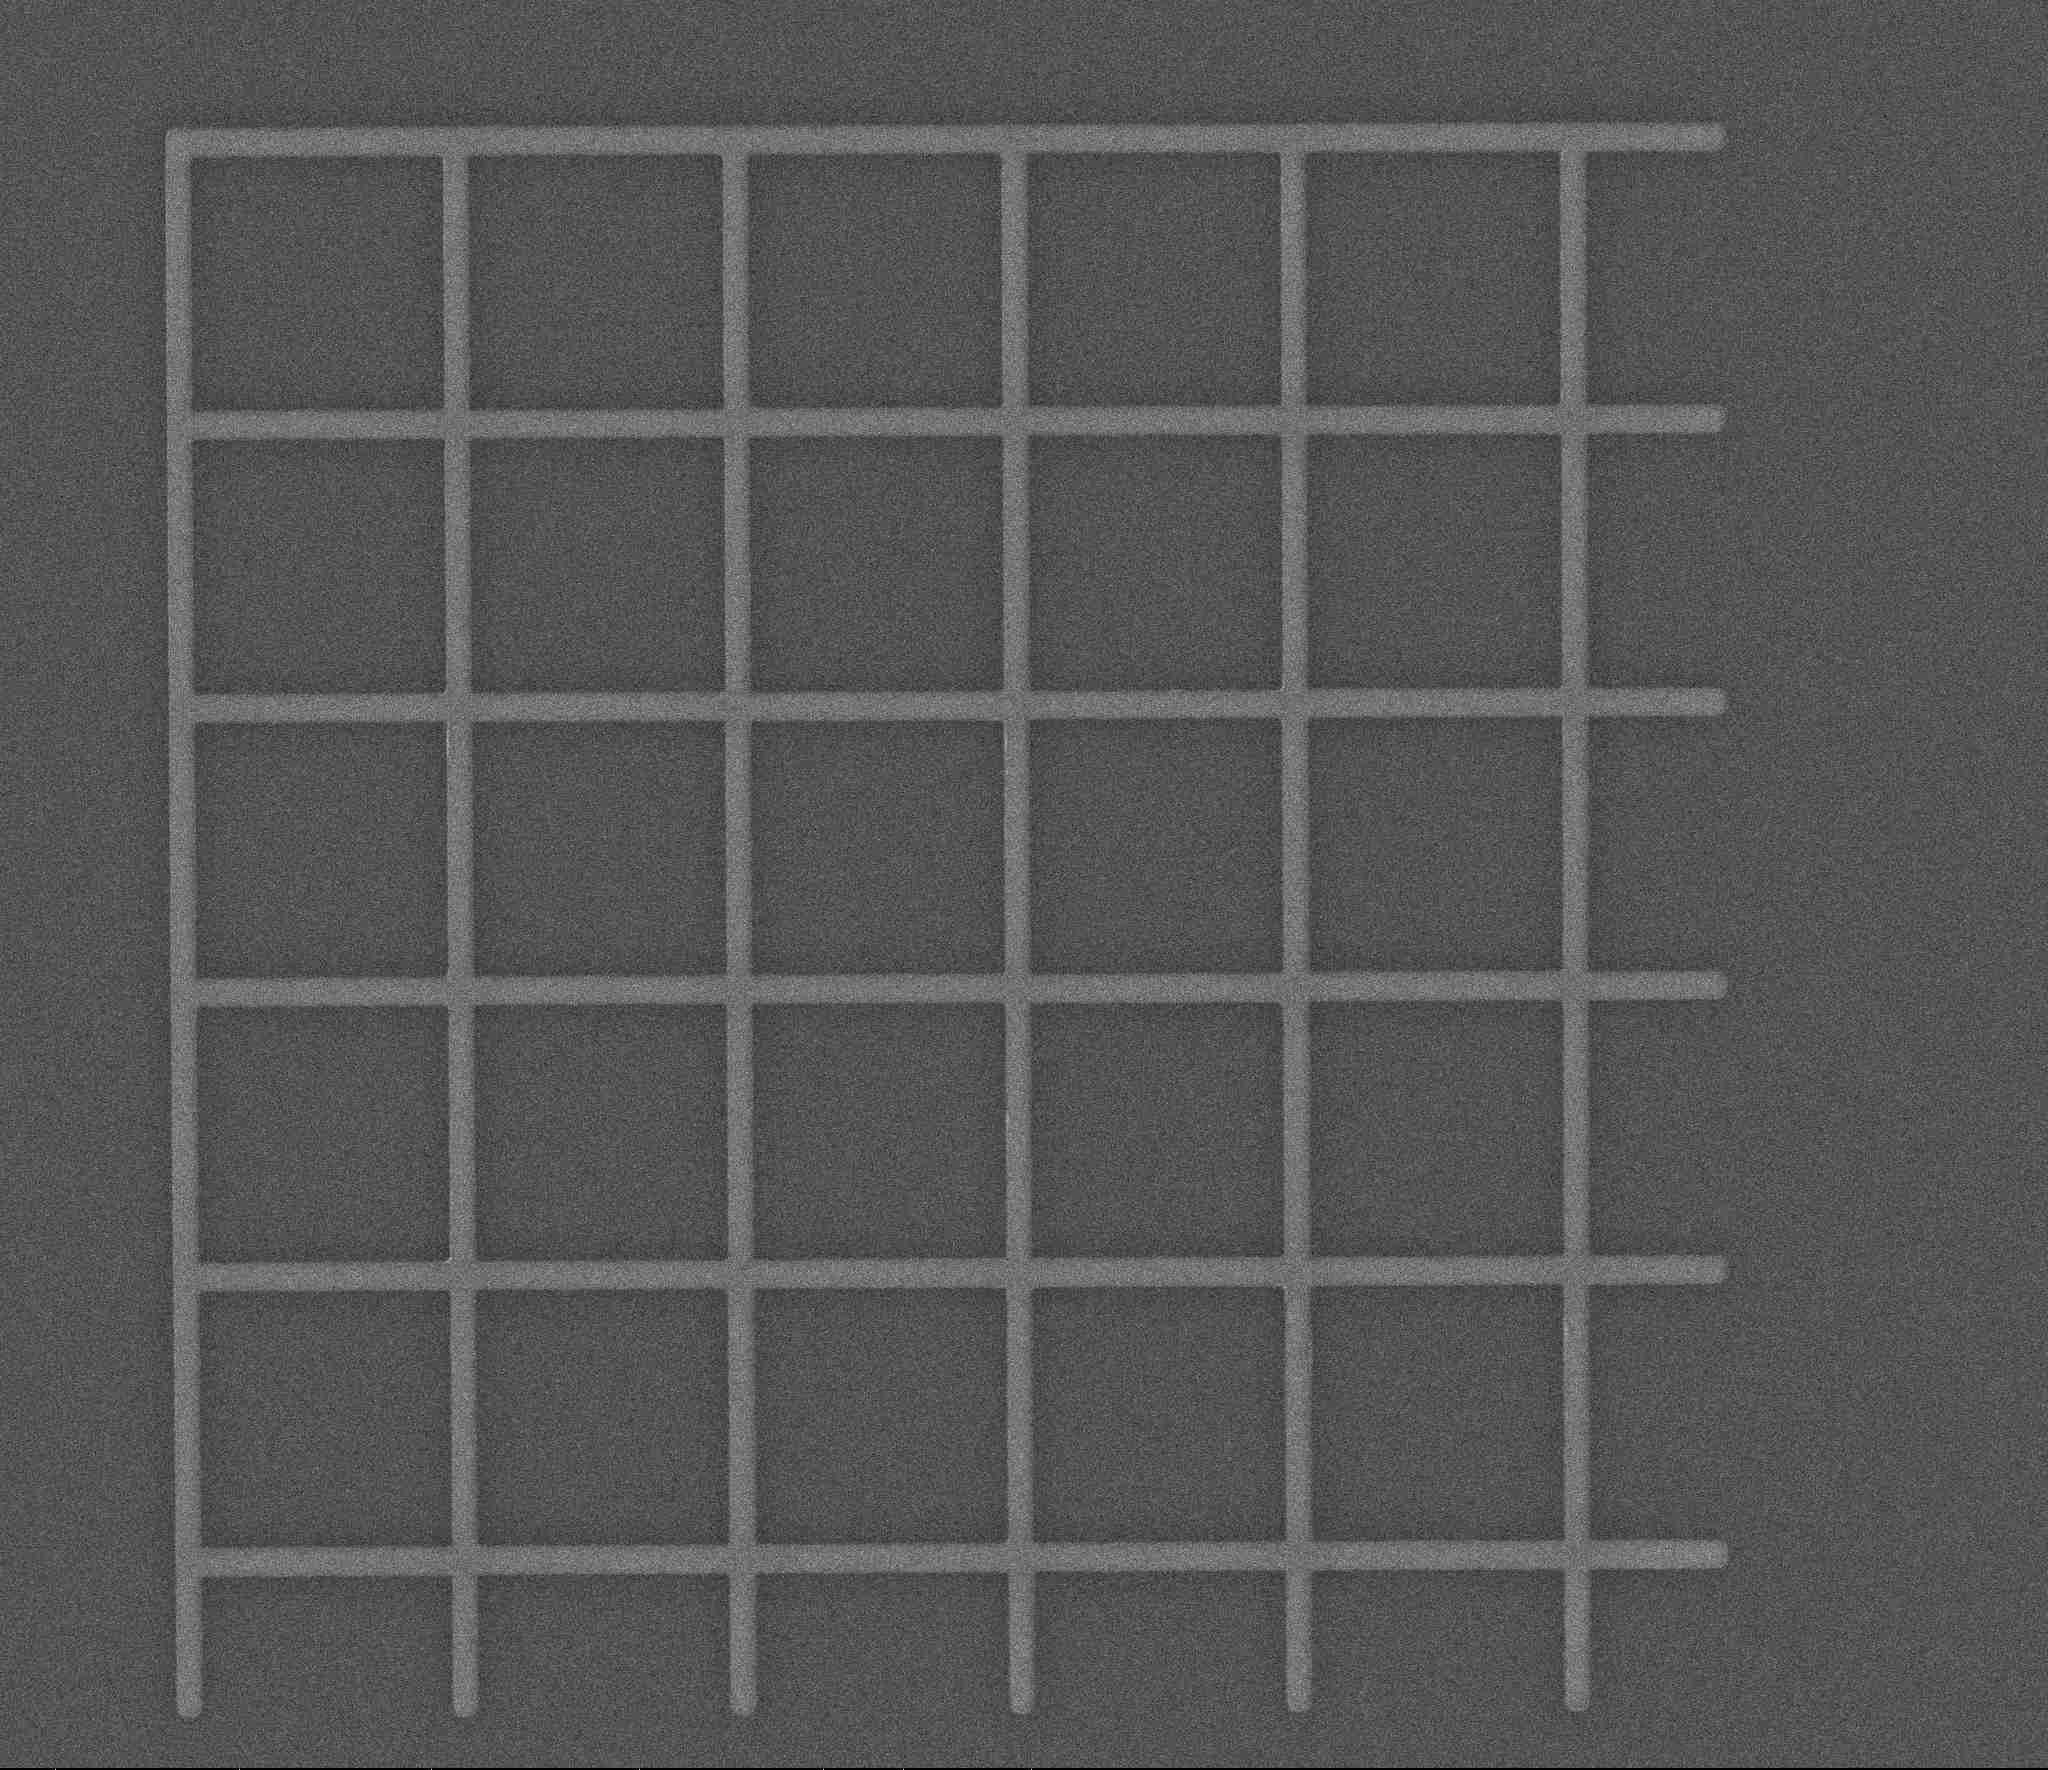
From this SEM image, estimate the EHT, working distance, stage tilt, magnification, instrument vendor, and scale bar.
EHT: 5 kV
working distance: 5.2 mm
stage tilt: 0°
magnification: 10 K X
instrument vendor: FEI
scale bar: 5000 nm